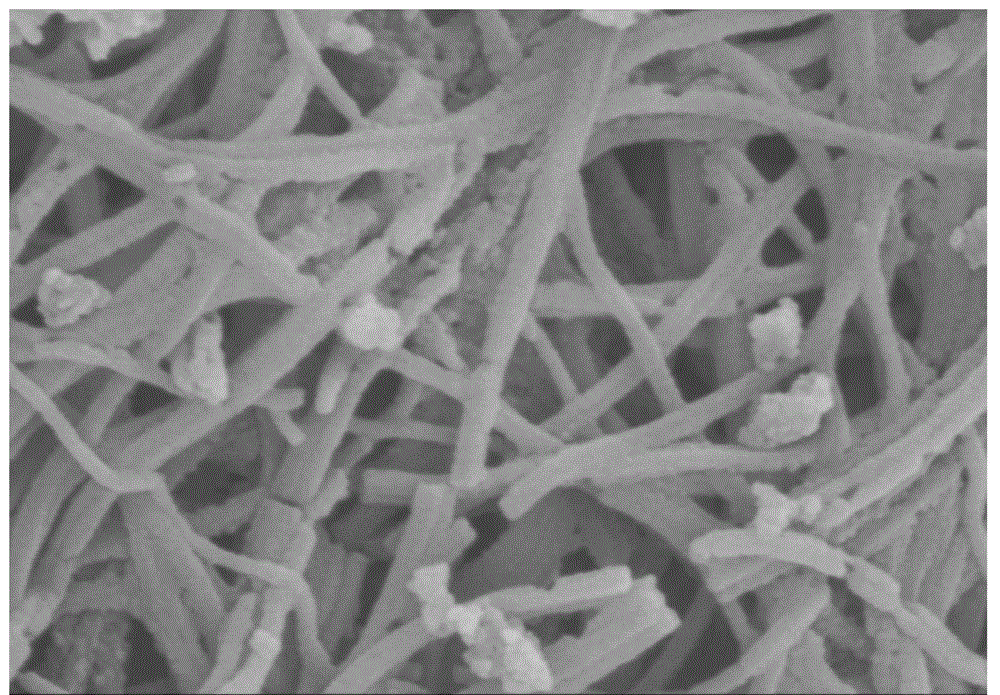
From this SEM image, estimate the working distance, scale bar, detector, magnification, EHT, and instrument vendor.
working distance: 9.6 mm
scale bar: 1000 nm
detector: SE(M)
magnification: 50 K X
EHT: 3 kV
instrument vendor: Hitachi